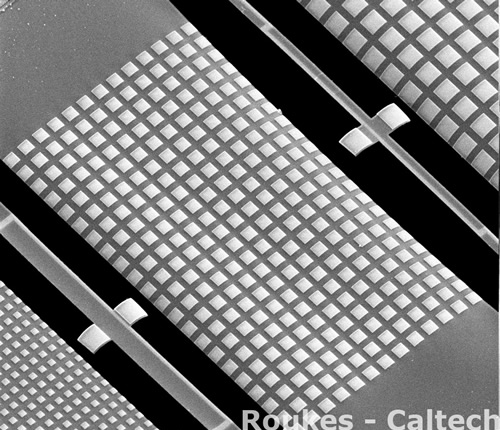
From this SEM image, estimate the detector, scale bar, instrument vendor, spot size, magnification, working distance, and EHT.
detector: ETD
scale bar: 20000 nm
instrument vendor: FEI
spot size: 2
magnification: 1.528 K X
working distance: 9 mm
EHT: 5 kV